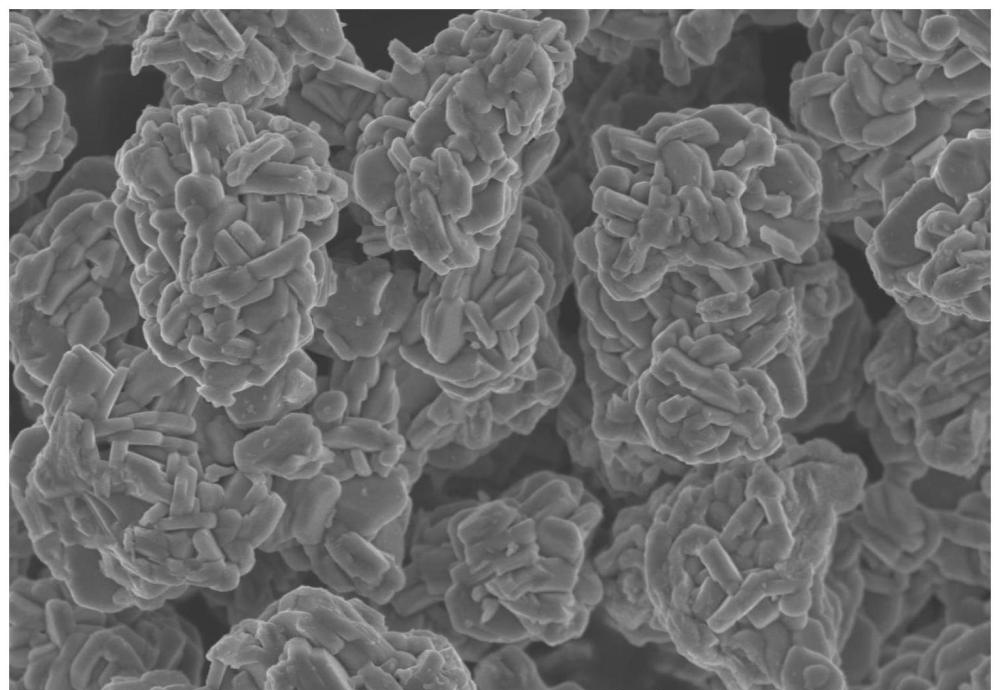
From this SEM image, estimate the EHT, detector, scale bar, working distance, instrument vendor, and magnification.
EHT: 5 kV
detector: SE(UL)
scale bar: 5000 nm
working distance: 8.8 mm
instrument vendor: Hitachi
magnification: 10 K X